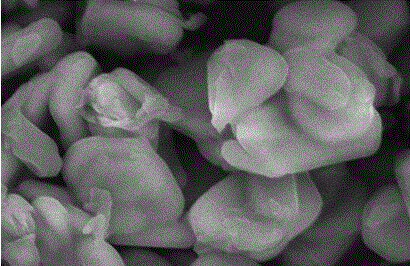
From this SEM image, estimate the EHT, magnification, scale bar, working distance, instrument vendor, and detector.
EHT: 10 kV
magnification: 17 K X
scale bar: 3000 nm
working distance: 5.7 mm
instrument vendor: Hitachi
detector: SE